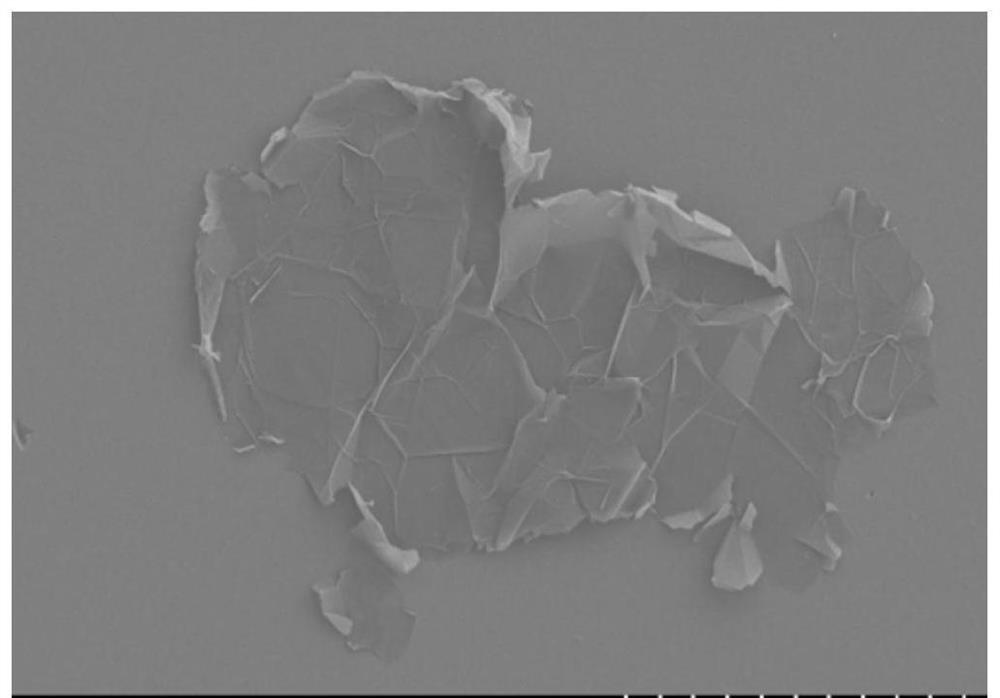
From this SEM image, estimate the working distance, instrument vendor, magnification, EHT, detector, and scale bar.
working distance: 18.1 mm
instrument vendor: Hitachi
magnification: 2.2 K X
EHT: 3 kV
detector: SE(L)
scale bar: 20000 nm